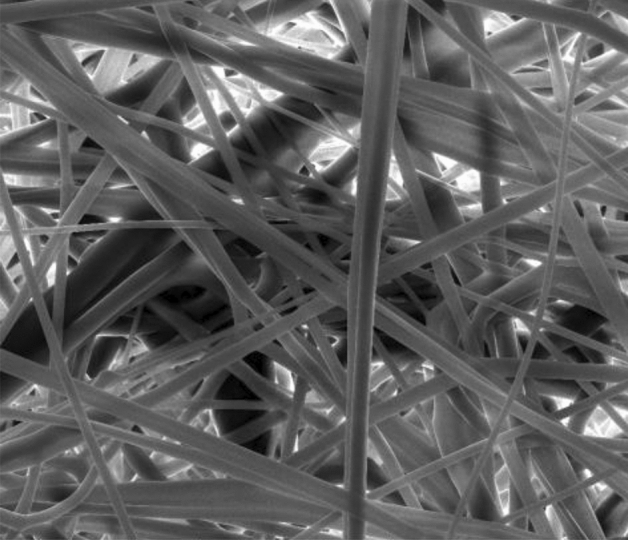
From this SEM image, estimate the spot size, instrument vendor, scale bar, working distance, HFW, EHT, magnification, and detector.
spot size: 3.5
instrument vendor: FEI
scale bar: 100000 nm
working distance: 14.7 mm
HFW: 298 µm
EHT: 25 kV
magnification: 1 K X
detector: ETD